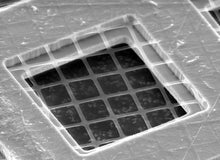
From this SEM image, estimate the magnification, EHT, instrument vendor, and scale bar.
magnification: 1.9 K X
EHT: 25 kV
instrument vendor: other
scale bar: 20000 nm